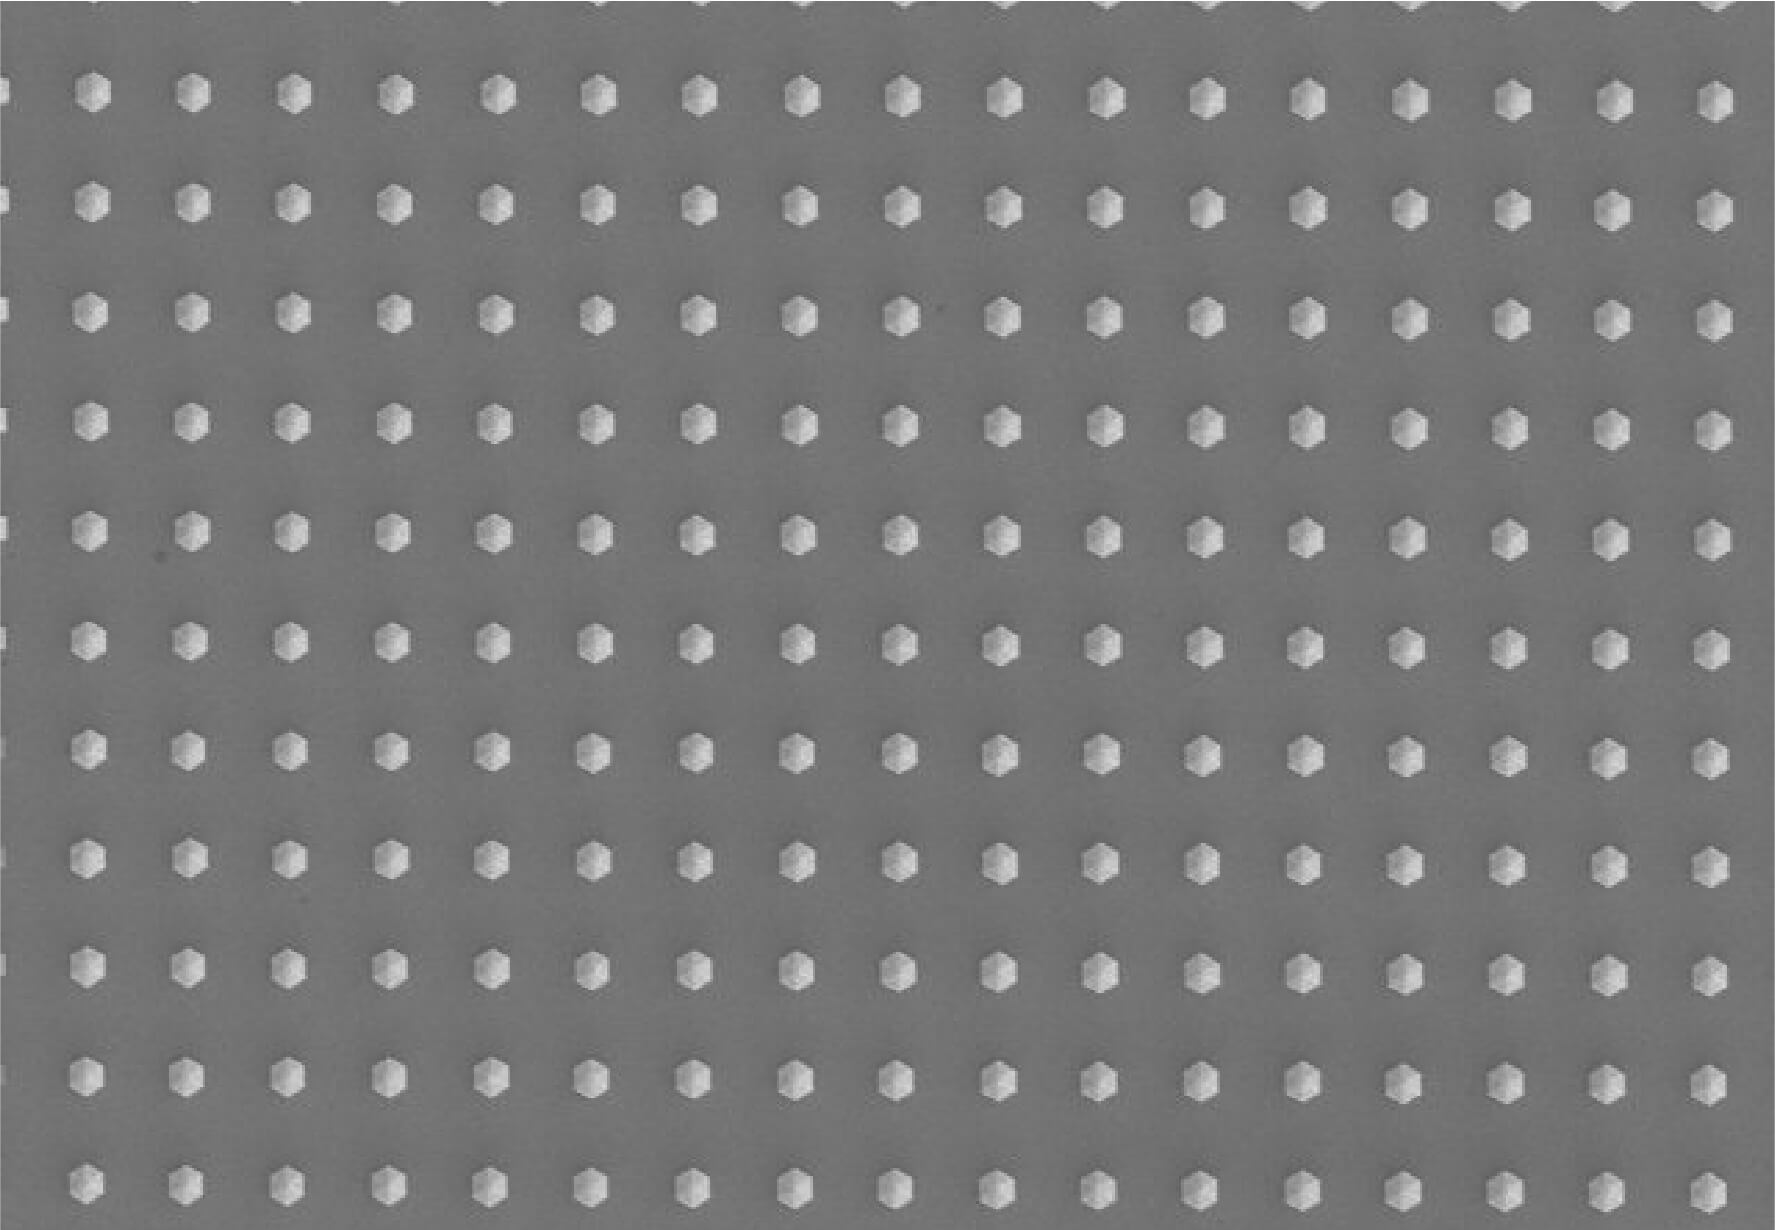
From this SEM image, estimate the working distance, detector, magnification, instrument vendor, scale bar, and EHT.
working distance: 13.5 mm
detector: SE(M)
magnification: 5 K X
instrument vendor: Hitachi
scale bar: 10000 nm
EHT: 3 kV